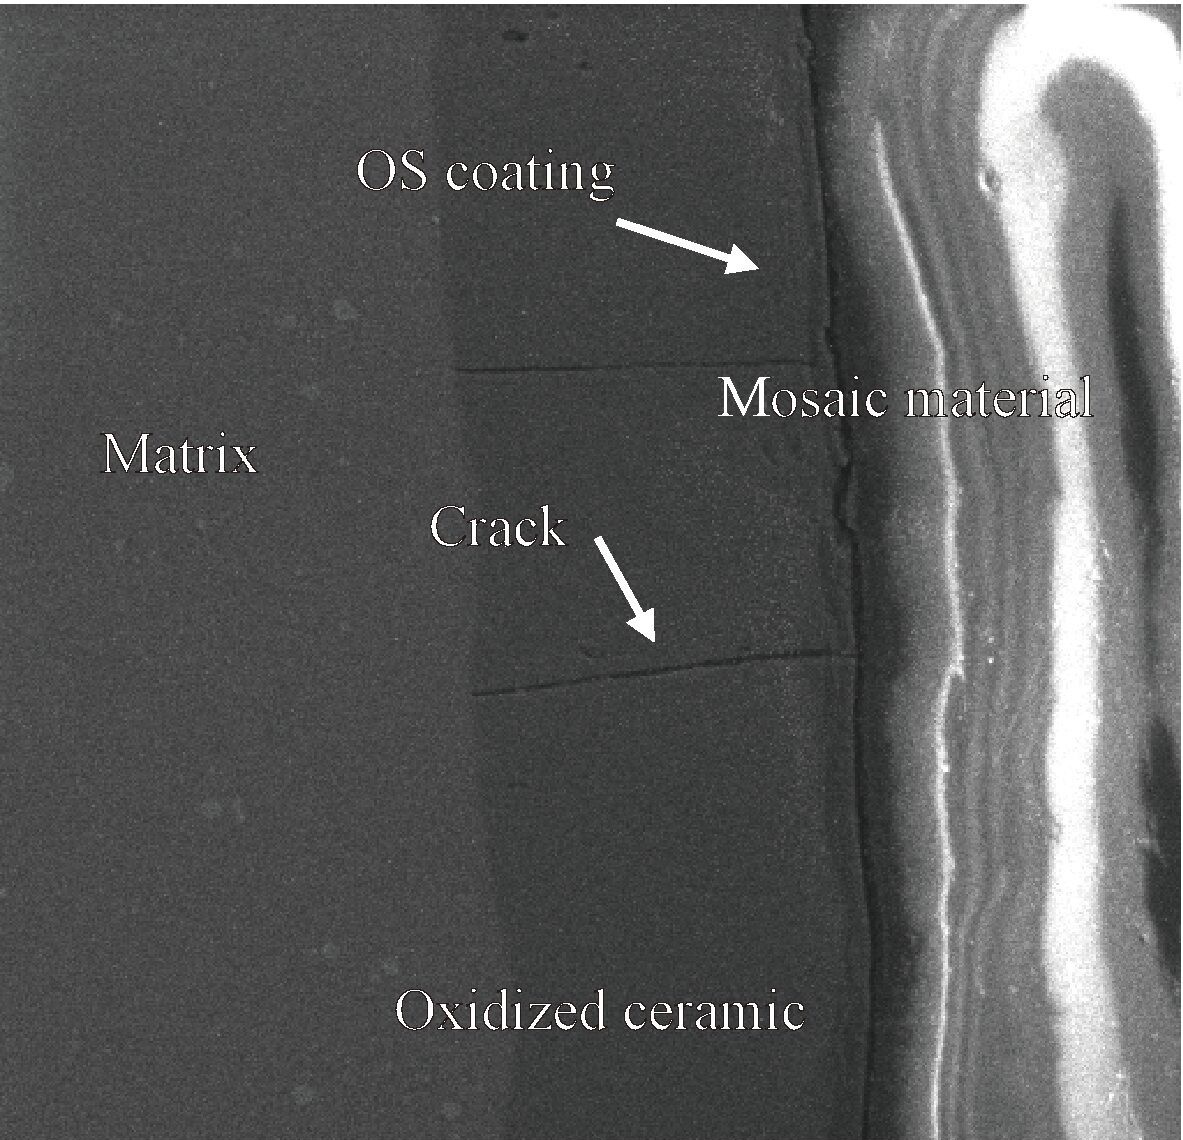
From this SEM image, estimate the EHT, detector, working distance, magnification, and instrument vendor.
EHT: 10 kV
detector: SE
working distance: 25.09 mm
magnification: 1 K X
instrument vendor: Tescan